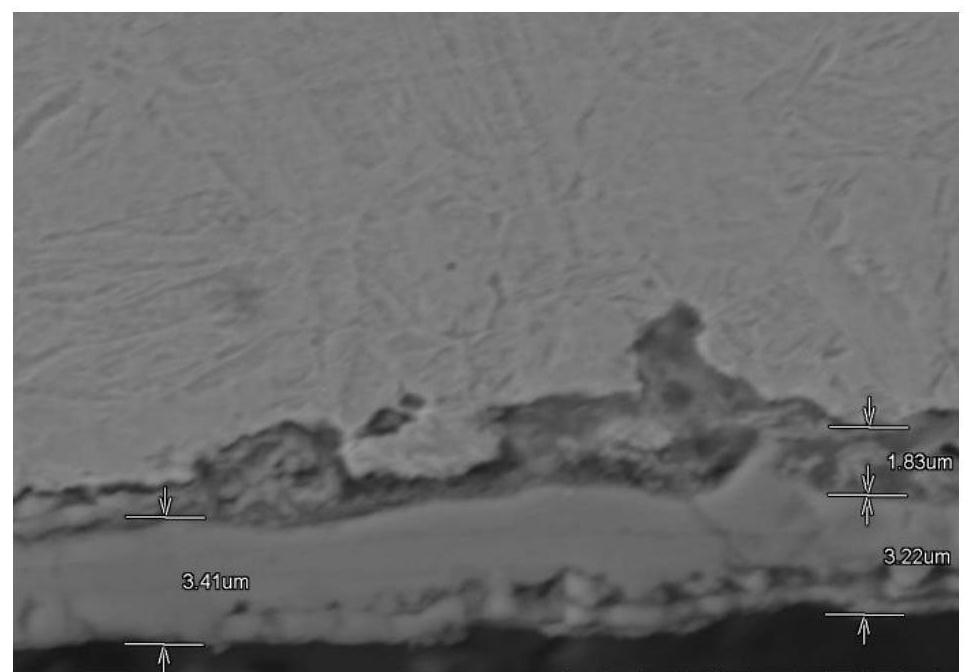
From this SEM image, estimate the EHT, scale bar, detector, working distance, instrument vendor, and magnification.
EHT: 15 kV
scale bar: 10000 nm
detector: BSE3D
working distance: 9.2 mm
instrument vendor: Hitachi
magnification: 5 K X